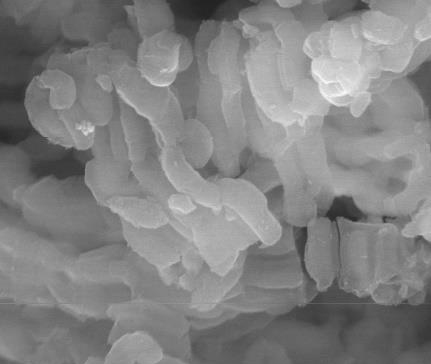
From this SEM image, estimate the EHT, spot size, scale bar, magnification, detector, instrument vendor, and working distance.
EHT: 30 kV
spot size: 4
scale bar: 2000 nm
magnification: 40 K X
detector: LFD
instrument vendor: FEI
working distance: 10 mm